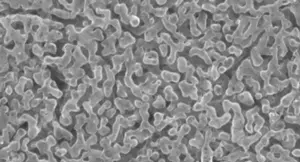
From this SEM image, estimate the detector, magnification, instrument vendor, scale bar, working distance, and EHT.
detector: SED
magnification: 8.5 K X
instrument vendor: JEOL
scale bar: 2000 nm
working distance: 13.1 mm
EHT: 10 kV